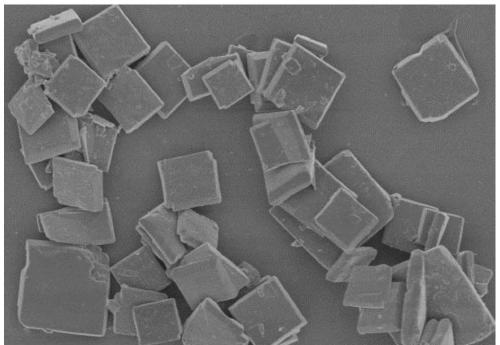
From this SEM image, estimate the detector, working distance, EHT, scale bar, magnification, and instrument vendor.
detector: SE(U)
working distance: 8.6 mm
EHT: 3 kV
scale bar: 20000 nm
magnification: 2 K X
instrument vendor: Hitachi